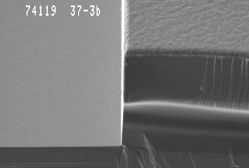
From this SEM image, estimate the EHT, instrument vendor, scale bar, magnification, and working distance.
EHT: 5 kV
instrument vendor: JEOL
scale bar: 10000 nm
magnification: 2 K X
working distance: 39 mm